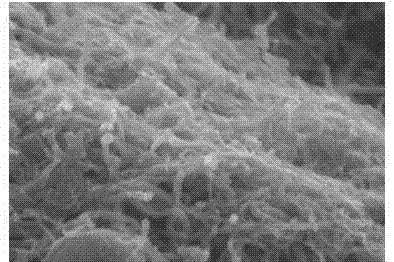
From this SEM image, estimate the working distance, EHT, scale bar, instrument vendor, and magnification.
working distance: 11.5 mm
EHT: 5 kV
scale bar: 1000 nm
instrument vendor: Hitachi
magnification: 40 K X